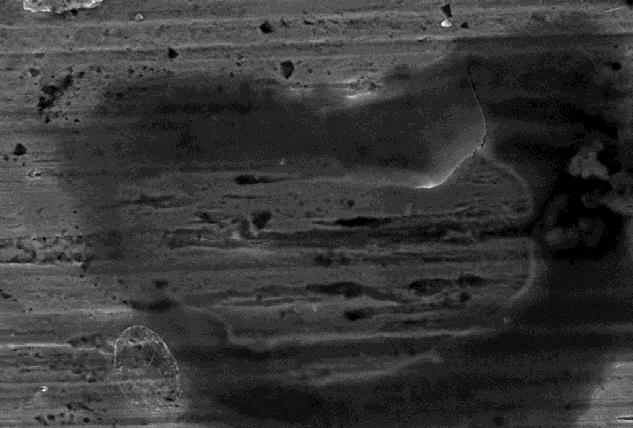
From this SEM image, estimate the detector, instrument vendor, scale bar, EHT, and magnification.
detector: SE1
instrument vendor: Zeiss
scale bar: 3000 nm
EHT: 15 kV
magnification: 5 K X